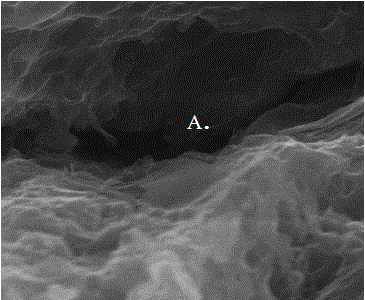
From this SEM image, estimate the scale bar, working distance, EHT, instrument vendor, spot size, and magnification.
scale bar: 3000 nm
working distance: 9.1 mm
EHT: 20 kV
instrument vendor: FEI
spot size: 2.5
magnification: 30 K X